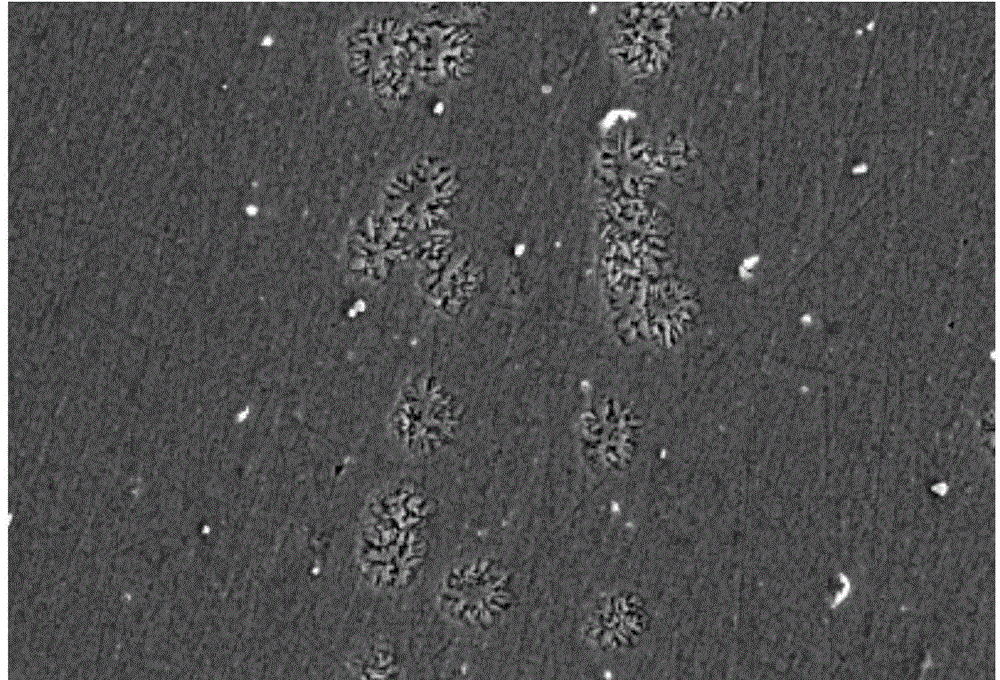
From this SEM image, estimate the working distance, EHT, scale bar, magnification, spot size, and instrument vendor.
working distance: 15 mm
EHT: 20 kV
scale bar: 10000 nm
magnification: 1 K X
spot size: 59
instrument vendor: JEOL